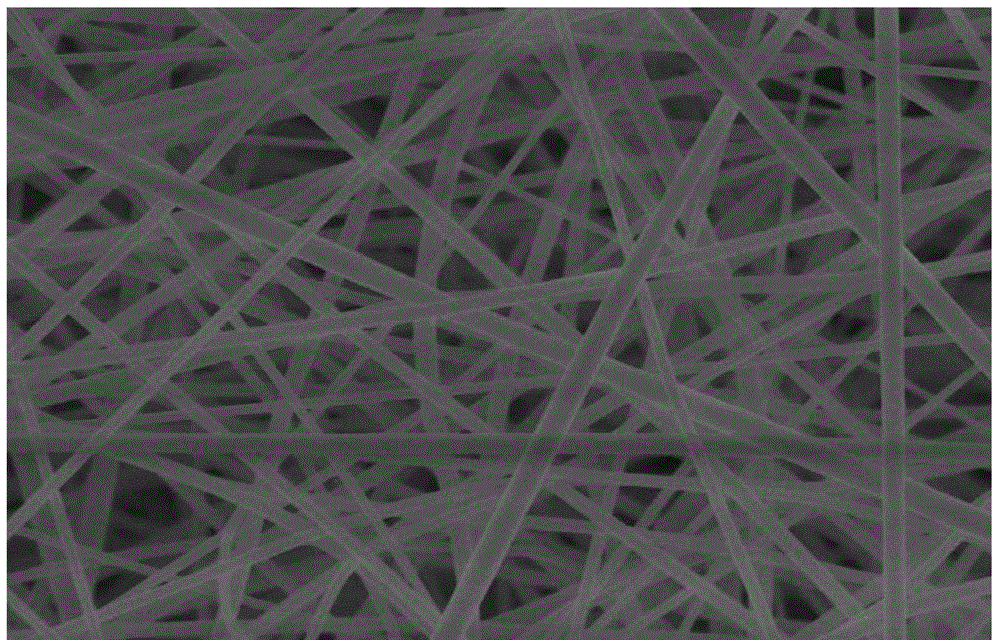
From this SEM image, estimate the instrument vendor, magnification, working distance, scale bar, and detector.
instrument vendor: Hitachi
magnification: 5 K X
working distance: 6.5 mm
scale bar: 10000 nm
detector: SE(M)